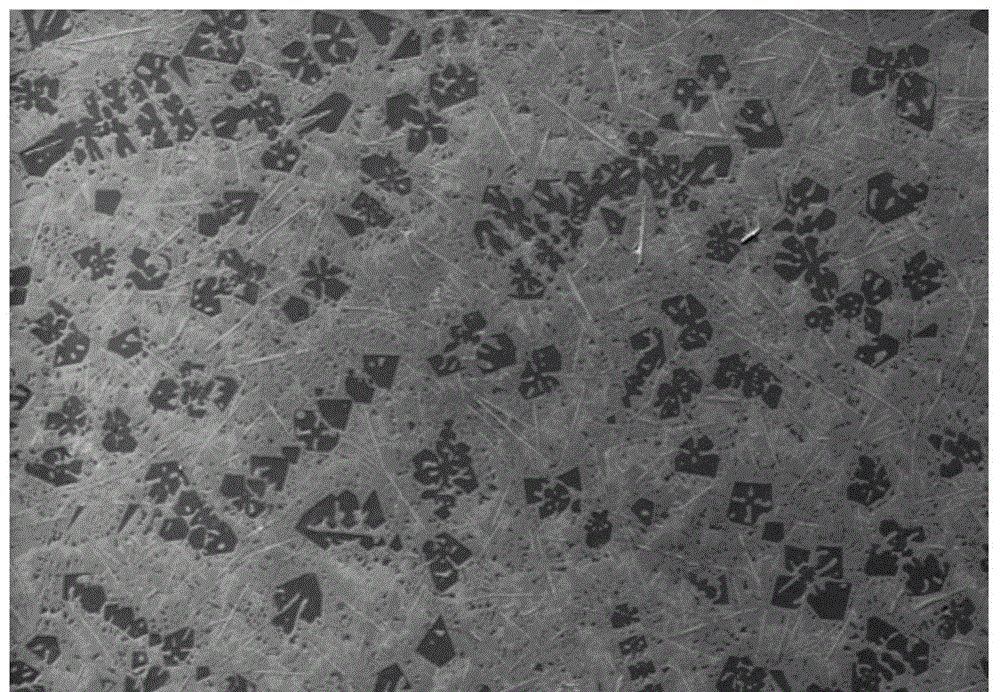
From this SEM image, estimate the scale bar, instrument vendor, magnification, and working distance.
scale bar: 30000 nm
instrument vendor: Zeiss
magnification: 0.1 K X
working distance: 10.5 mm